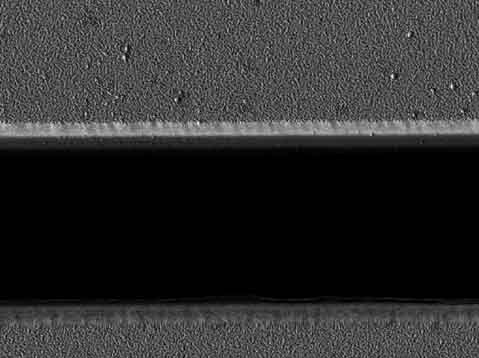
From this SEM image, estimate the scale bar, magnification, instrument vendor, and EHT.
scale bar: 1000 nm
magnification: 7 K X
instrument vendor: JEOL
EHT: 3 kV